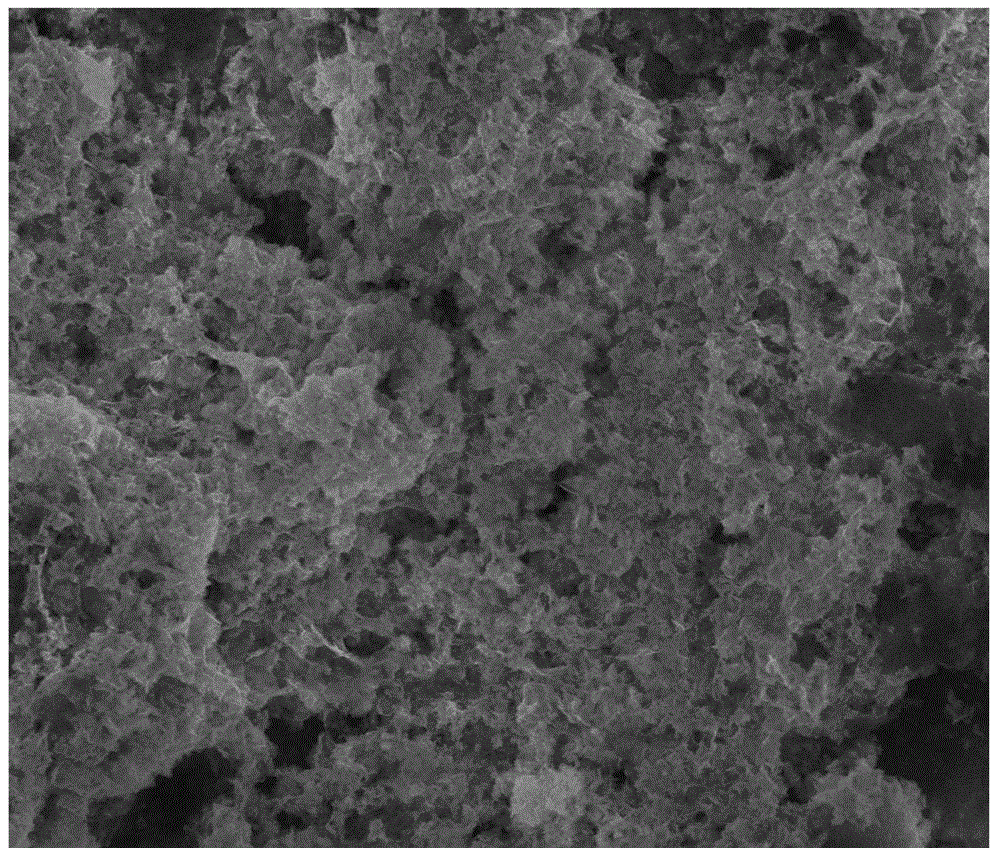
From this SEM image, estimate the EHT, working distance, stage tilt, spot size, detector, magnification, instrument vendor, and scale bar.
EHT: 15 kV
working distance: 5.5 mm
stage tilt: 0°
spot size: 3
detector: SE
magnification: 10 K X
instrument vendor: FEI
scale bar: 5000 nm